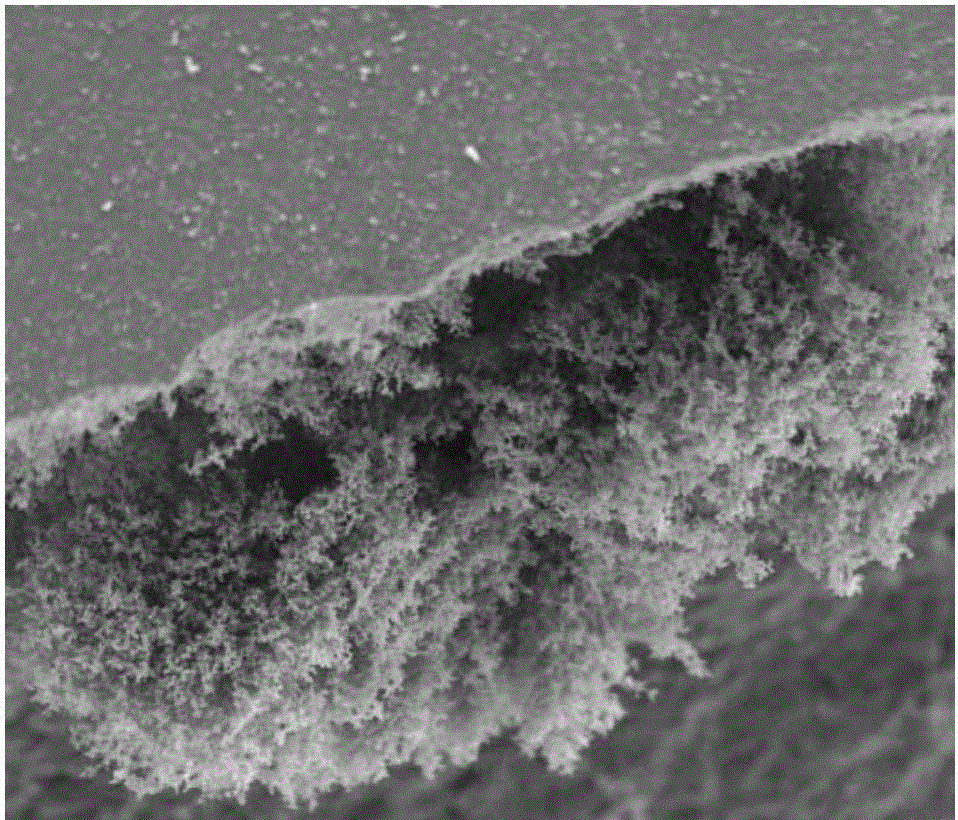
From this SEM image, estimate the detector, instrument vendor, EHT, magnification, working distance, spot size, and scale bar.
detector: ETD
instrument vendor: FEI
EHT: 15 kV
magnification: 0.3 K X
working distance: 10.7 mm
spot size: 6.5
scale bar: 100000 nm